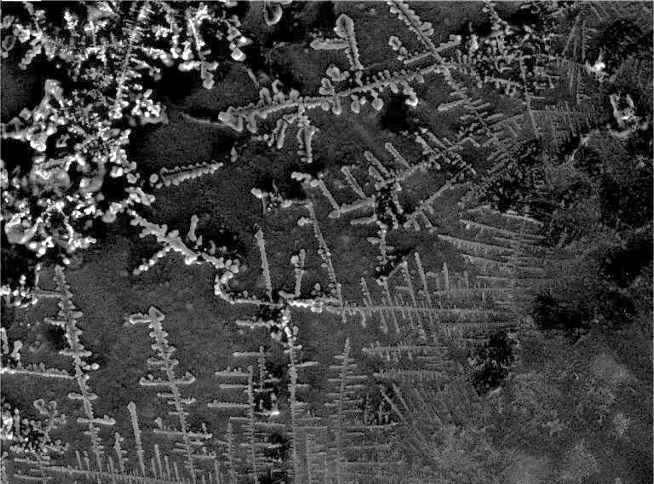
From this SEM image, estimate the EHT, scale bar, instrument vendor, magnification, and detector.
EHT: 30 kV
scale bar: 200000 nm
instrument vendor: Tescan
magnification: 0.169 K X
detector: SE Detector